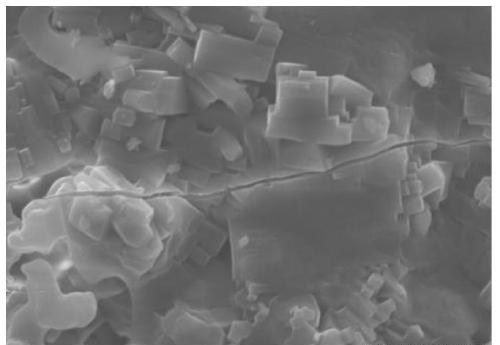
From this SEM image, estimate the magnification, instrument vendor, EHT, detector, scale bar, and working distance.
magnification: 3 K X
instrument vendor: Hitachi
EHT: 20 kV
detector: SE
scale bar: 10000 nm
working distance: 9.8 mm